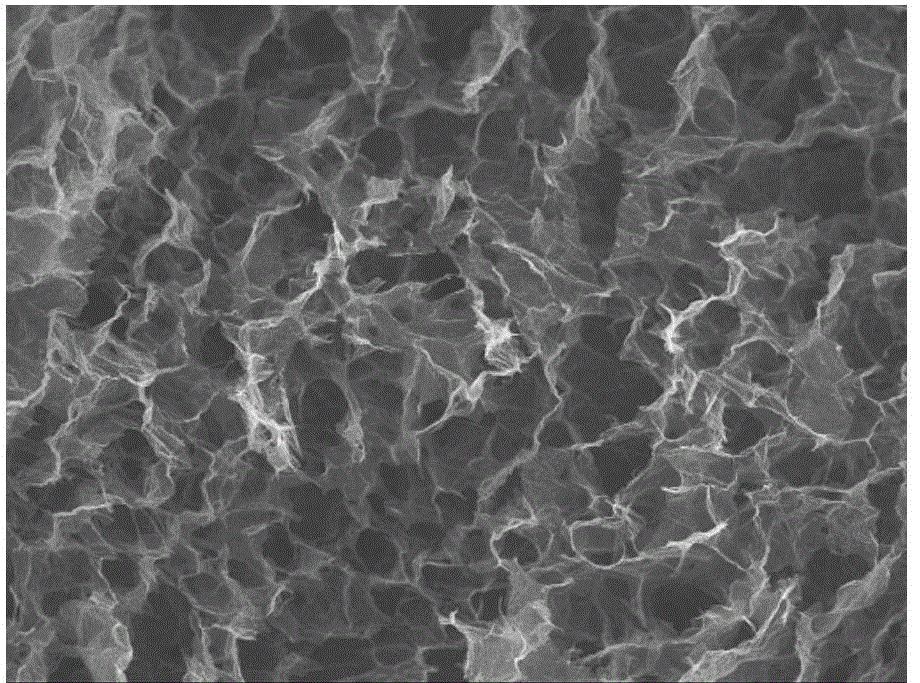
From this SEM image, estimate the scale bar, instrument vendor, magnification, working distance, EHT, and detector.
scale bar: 100000 nm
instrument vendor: JEOL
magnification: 0.2 K X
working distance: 9.8 mm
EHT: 10 kV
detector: SEI